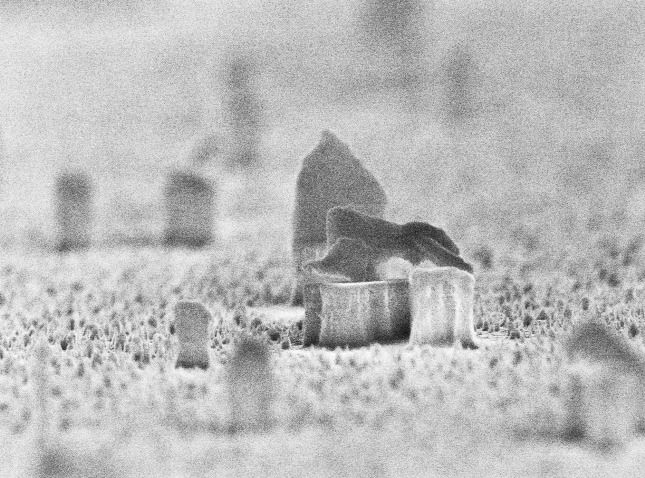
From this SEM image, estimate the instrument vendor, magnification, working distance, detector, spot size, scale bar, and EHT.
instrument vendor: FEI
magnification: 24.008 K X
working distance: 5.4 mm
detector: TLD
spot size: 2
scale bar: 1000 nm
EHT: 10 kV